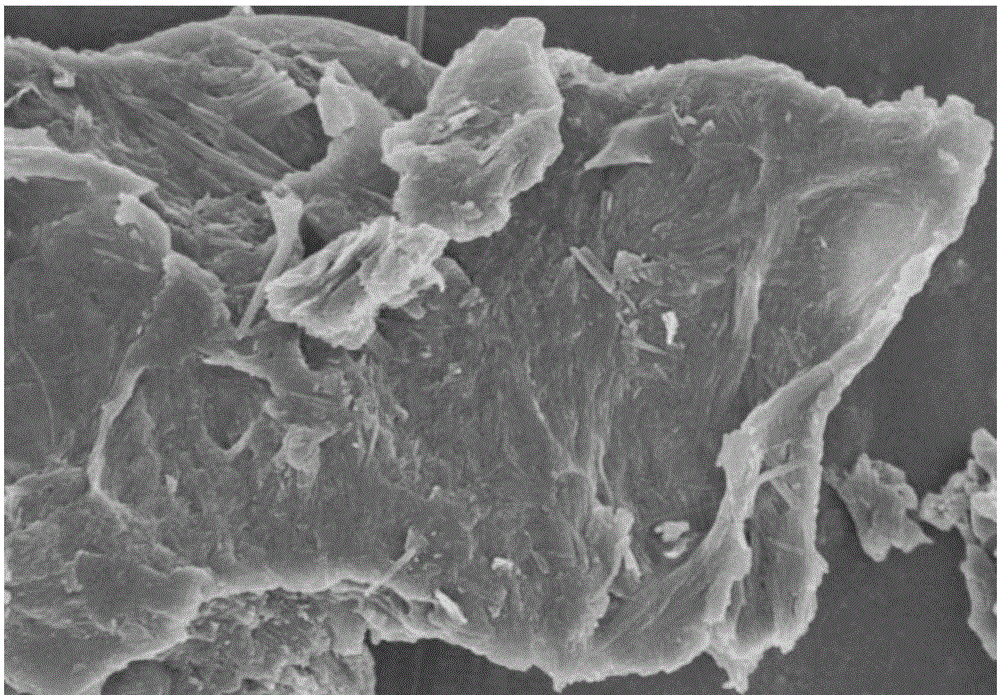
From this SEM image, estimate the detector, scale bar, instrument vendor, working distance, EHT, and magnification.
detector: SE(M)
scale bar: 2000 nm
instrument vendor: Hitachi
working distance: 8 mm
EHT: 3 kV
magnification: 20 K X